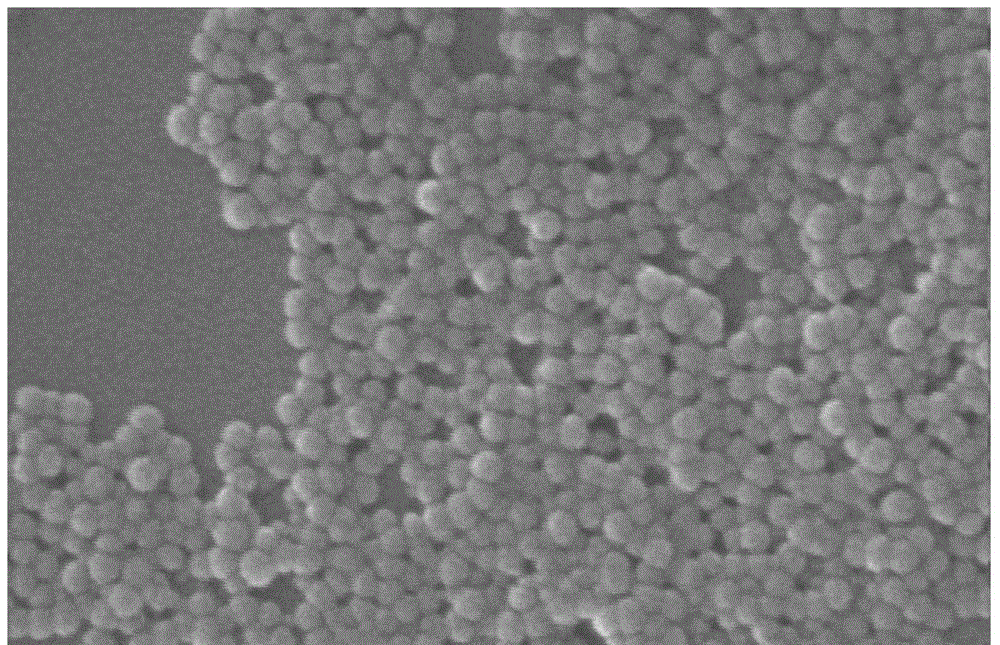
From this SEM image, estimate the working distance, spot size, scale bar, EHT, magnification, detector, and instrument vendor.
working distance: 11 mm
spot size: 3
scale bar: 200 nm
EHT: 25 kV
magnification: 80 K X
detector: SE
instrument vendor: FEI